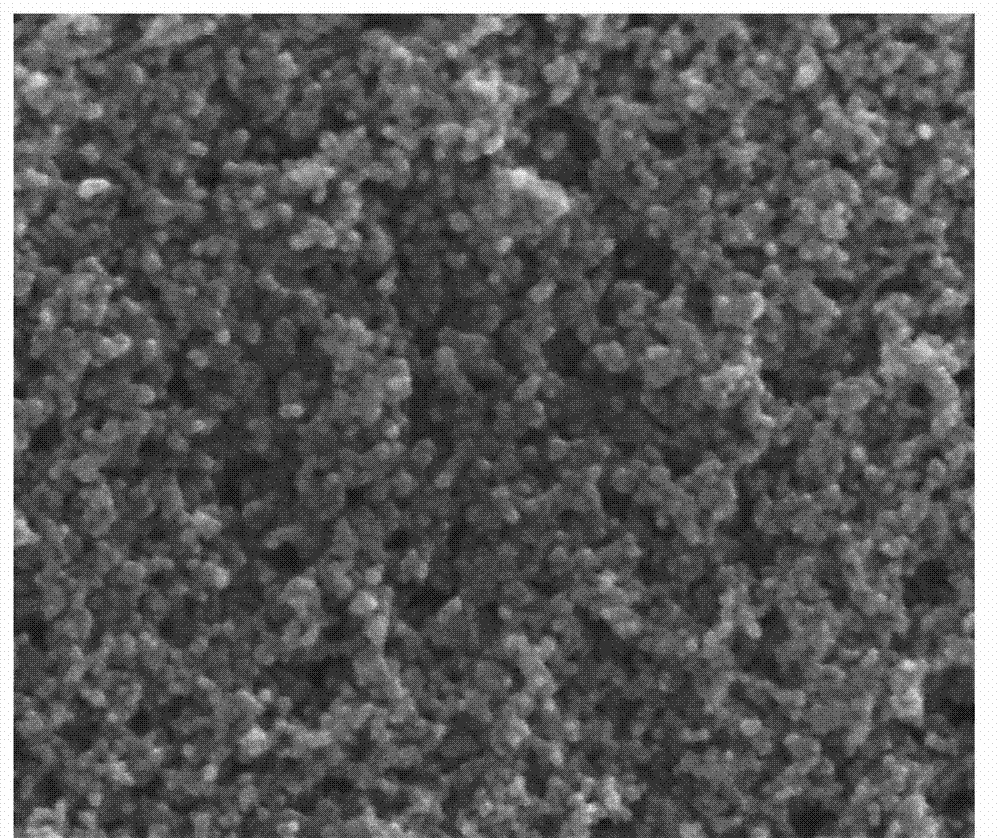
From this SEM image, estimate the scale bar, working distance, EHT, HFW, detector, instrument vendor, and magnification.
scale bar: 400 nm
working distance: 6.3 mm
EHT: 5 kV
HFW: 1.46 µm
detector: TLD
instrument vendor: FEI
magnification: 203.76 K X